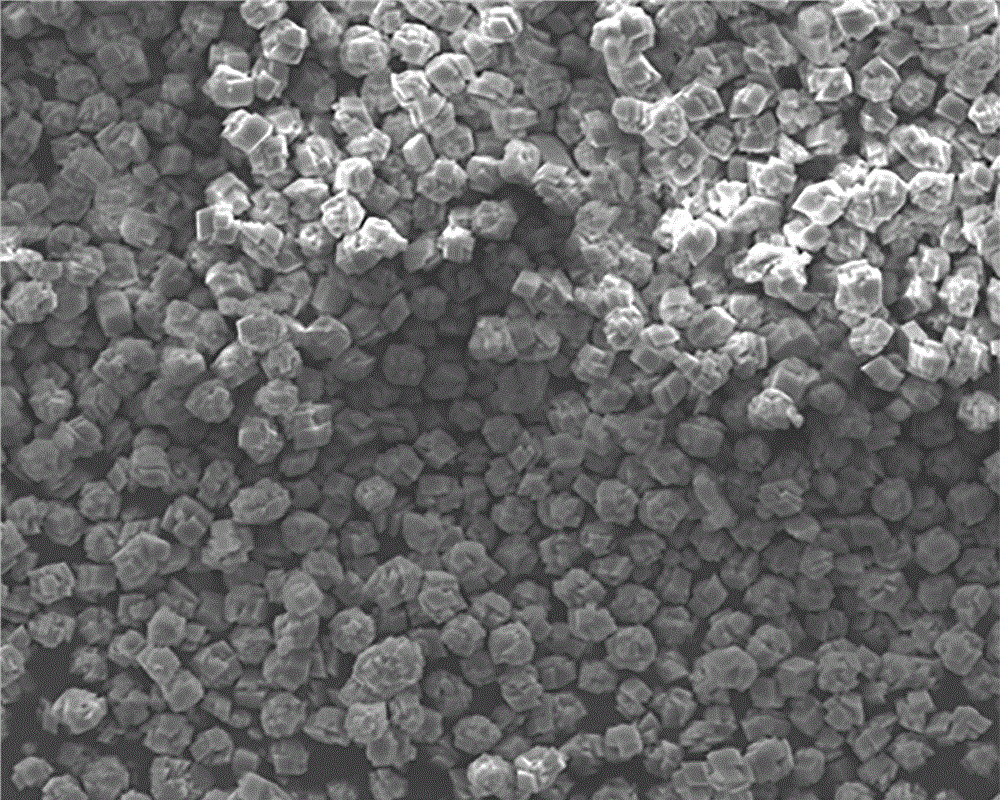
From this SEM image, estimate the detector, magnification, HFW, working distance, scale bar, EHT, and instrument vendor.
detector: ETD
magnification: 1 K X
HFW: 140 µm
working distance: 8.8 mm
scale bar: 20000 nm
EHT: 20 kV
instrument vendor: FEI